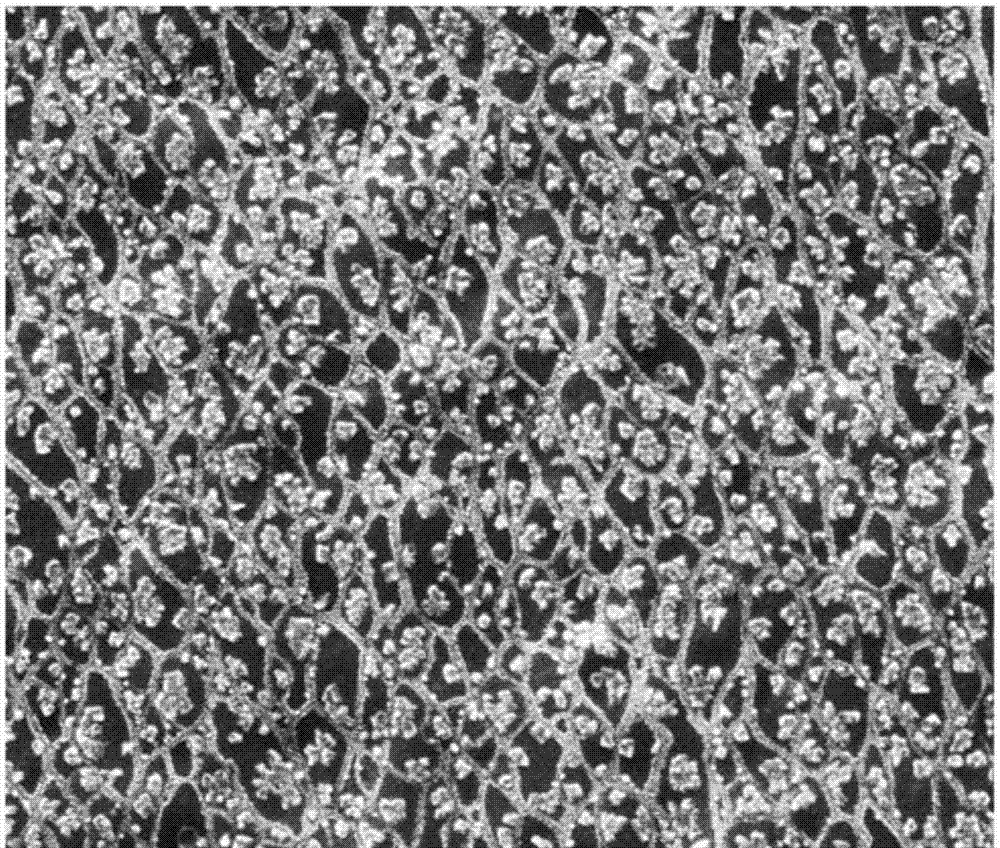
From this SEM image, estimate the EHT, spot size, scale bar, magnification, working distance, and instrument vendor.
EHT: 15 kV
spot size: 3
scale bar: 20000 nm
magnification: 4 K X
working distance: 26.5 mm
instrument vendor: FEI